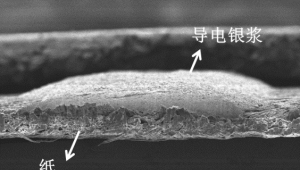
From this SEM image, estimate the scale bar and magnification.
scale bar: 100000 nm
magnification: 0.12 K X